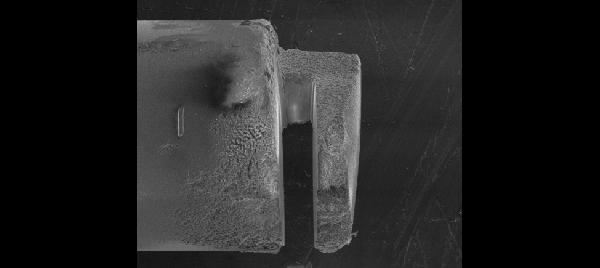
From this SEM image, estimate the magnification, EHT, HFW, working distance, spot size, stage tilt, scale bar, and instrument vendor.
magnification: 0.847 K X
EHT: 5 kV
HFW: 176 µm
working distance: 10 mm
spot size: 4.5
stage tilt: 0°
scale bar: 50000 nm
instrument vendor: FEI